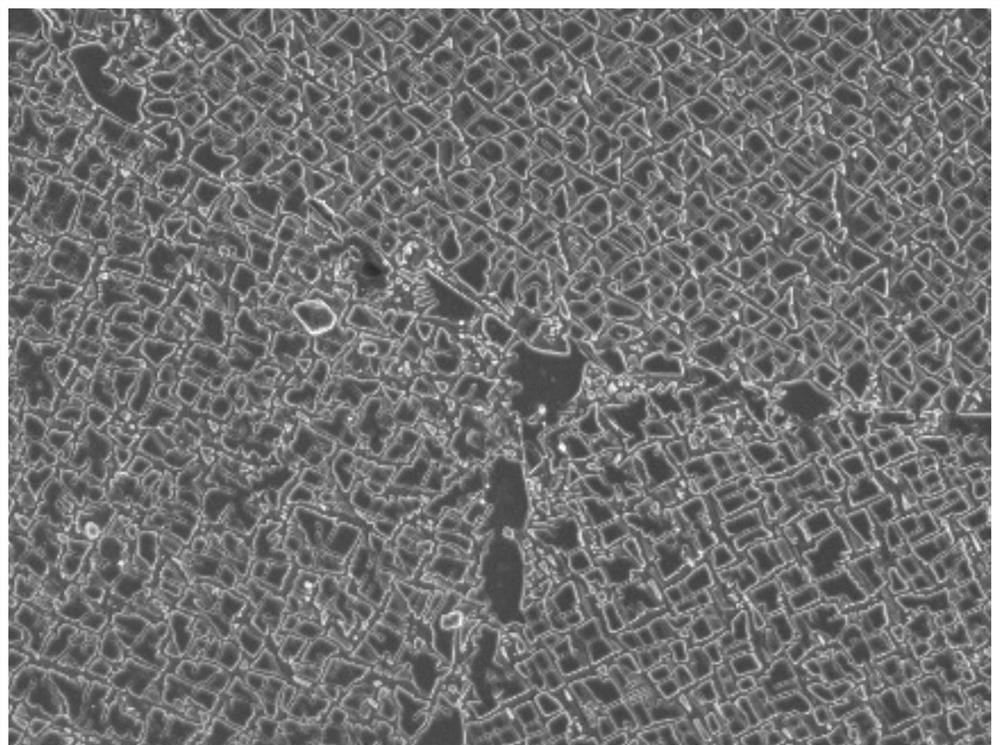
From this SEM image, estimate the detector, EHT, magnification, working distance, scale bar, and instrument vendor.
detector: SEI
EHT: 5 kV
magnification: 5 K X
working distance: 8.3 mm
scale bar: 1000 nm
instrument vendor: JEOL